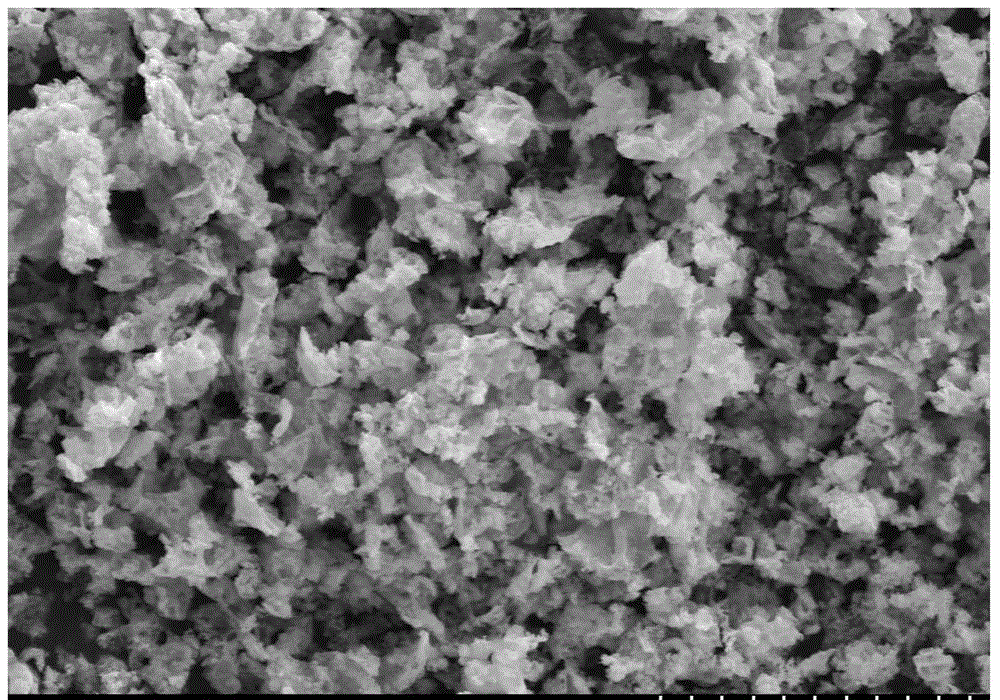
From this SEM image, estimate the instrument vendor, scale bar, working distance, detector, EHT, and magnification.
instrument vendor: Hitachi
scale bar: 20000 nm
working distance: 15.4 mm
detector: SE(M)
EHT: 15 kV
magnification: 2 K X